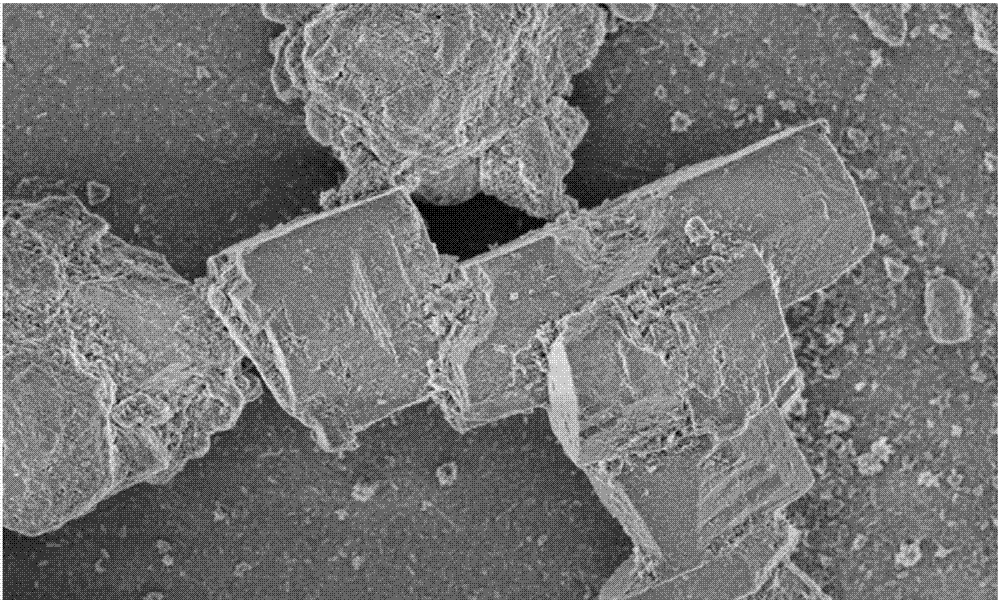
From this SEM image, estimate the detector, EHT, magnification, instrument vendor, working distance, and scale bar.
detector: SE(U)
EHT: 2 kV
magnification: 5 K X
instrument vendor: Hitachi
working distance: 8.5 mm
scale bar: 10000 nm